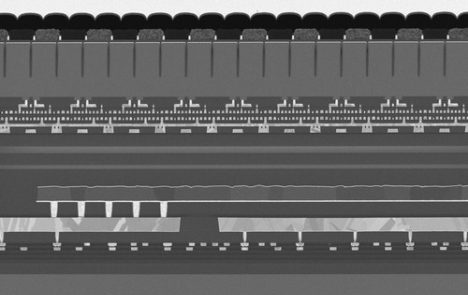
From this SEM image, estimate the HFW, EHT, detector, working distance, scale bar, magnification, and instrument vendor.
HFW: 25.4 µm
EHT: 1.5 kV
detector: TLD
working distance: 2 mm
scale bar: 10000 nm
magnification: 5 K X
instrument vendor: Thermo